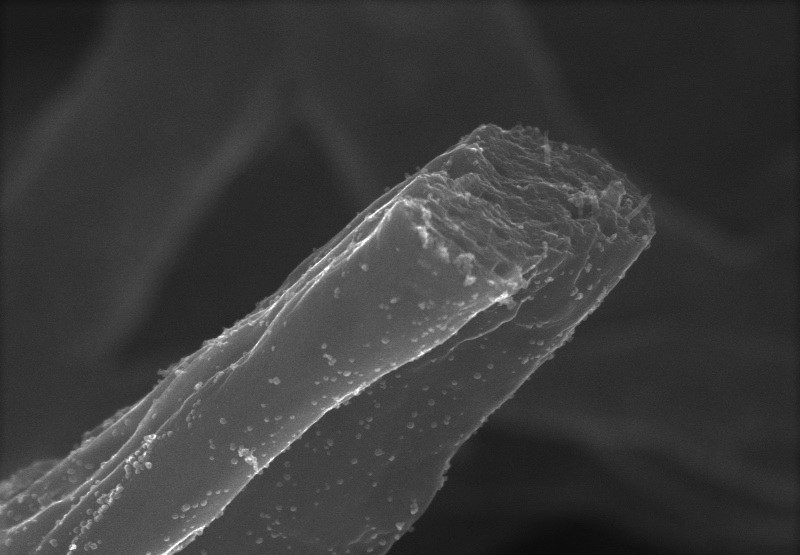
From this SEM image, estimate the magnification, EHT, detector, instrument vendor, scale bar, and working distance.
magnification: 50 K X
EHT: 15 kV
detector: SE(UL)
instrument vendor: Hitachi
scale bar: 1000 nm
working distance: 6.3 mm